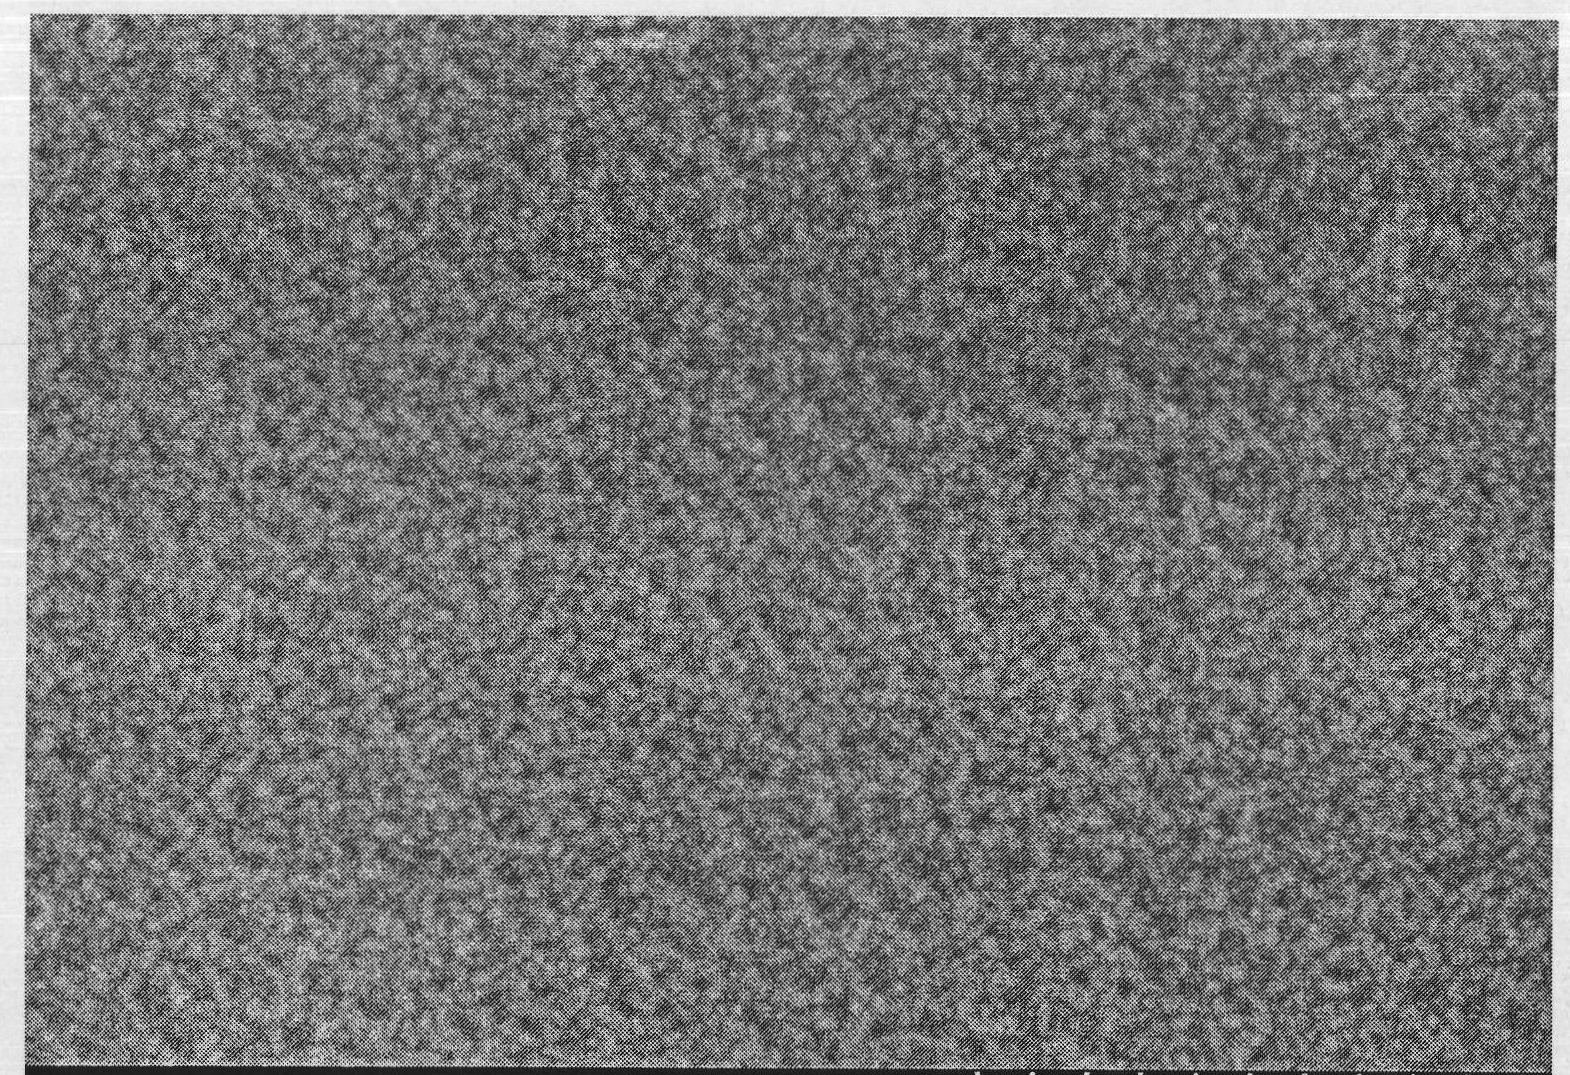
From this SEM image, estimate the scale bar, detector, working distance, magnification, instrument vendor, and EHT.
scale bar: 300 nm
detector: SE(M)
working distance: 13 mm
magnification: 150 K X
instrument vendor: Hitachi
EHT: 10 kV